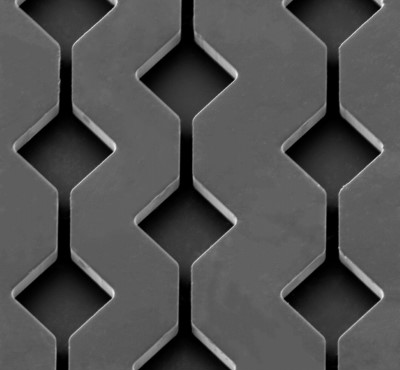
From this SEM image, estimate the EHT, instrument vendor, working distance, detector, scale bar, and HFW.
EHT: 10 kV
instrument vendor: Tescan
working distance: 16.43 mm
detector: SE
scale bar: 100000 nm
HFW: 610 µm